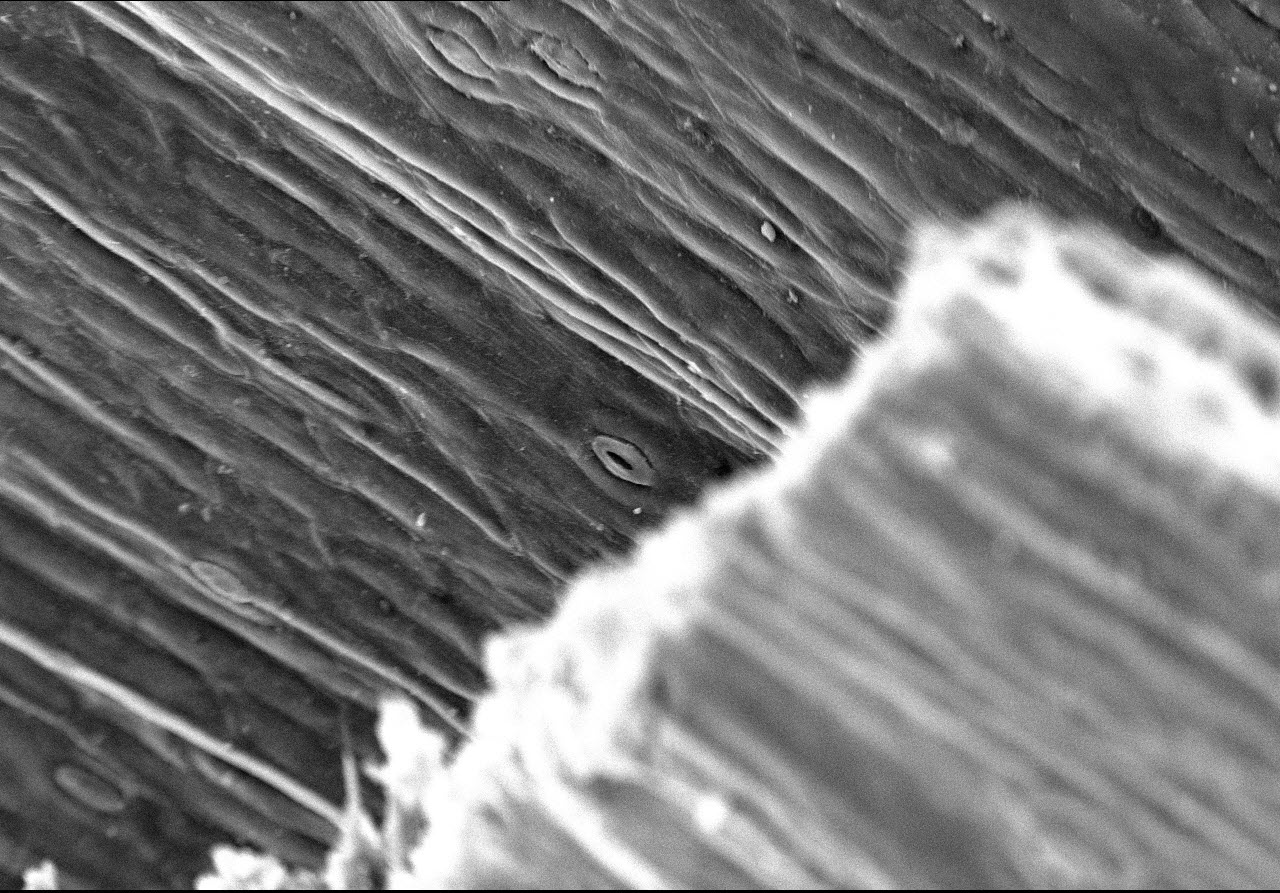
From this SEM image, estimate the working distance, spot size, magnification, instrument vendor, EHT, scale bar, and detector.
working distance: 11.9 mm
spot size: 10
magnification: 0.5 K X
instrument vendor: other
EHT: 20 kV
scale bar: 100000 nm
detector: SEI